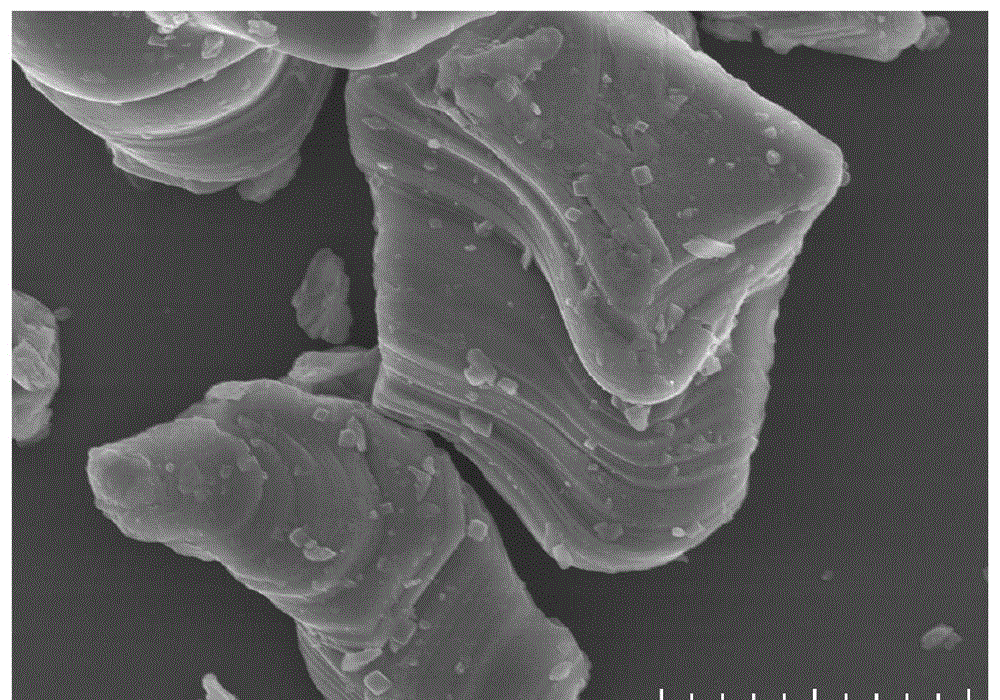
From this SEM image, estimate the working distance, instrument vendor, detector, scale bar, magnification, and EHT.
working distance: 8.3 mm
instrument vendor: Hitachi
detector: SE(UL)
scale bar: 5000 nm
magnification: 8 K X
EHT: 10 kV